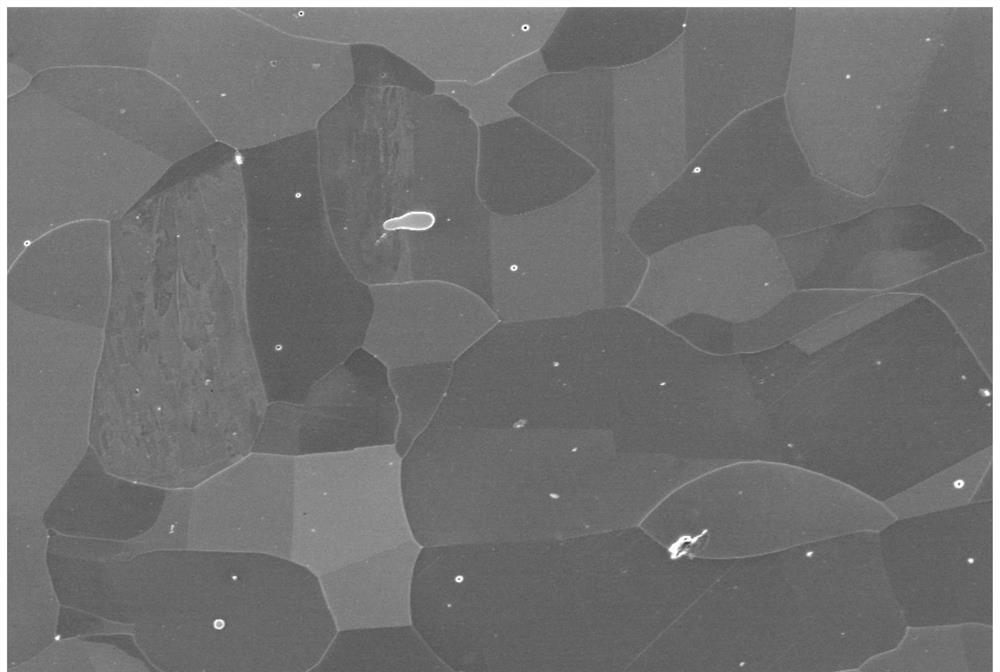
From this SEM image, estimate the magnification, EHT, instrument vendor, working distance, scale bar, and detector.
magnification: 3 K X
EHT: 5 kV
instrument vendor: Zeiss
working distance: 8.9 mm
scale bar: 2000 nm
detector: InLens